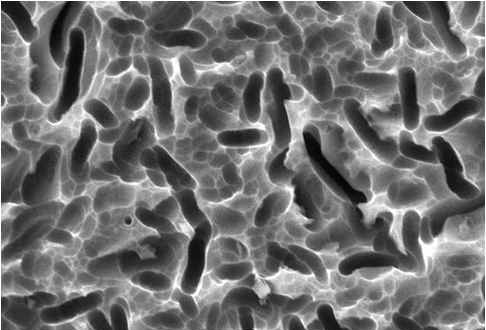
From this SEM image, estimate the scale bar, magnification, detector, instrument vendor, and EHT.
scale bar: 5000 nm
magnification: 3 K X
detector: SEI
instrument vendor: JEOL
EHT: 20 kV